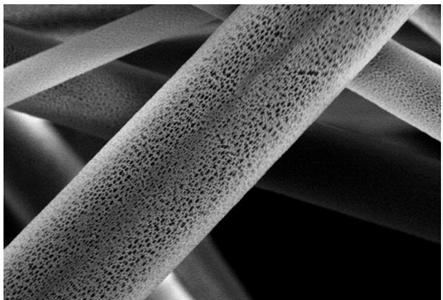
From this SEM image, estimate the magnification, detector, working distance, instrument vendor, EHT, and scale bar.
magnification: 3 K X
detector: SE(M)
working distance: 7.9 mm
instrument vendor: Hitachi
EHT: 15 kV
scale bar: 10000 nm